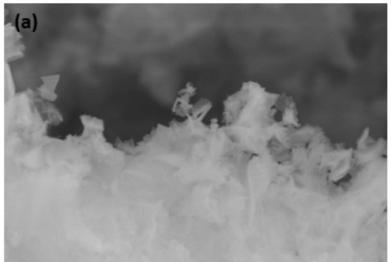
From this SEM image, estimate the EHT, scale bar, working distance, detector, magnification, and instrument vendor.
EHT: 15 kV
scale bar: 4000 nm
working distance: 17.6 mm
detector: SE(M)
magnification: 13 K X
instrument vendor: Hitachi